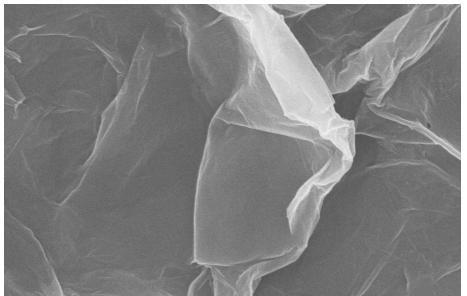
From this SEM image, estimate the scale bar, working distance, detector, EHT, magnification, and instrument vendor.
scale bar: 2000 nm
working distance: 8.4 mm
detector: SE(U)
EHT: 10 kV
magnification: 20 K X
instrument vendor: Hitachi